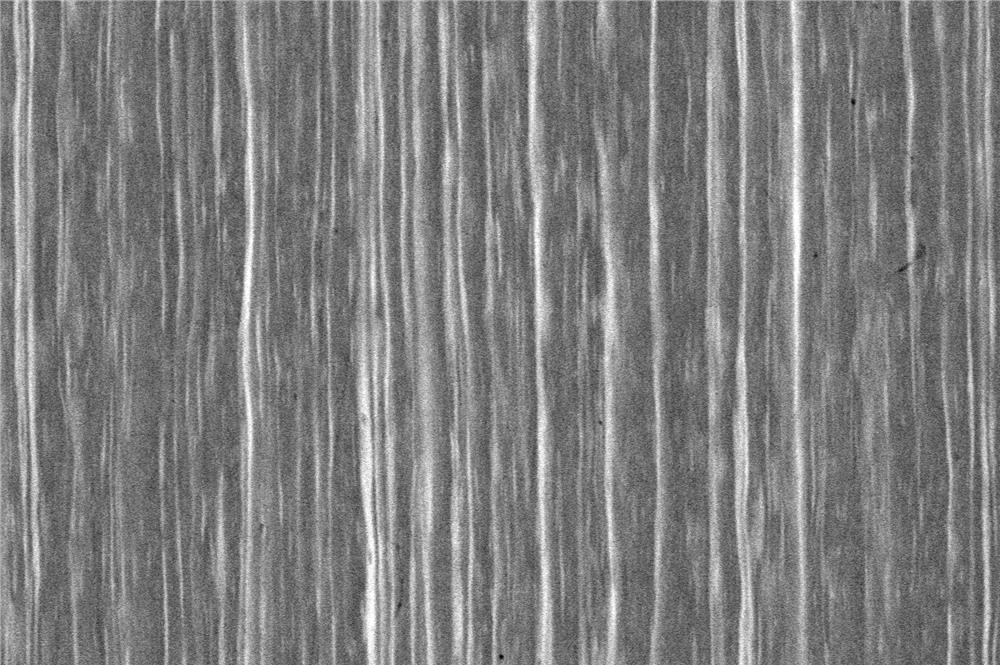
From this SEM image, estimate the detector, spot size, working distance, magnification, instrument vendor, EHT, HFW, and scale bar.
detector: BSED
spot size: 3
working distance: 14.5 mm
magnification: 15 K X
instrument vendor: FEI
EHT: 20 kV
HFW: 27.6 µm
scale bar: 10000 nm